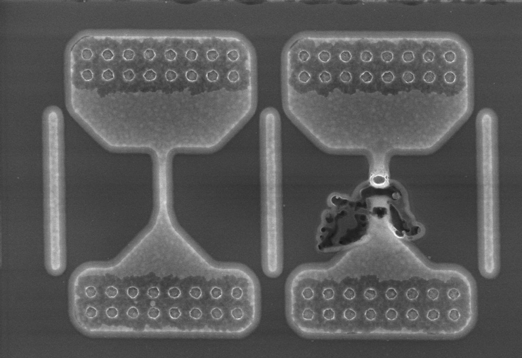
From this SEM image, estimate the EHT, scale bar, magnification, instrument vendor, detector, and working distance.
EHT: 10 kV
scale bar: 3000 nm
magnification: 15 K X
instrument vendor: Hitachi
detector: SE(M)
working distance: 9.1 mm